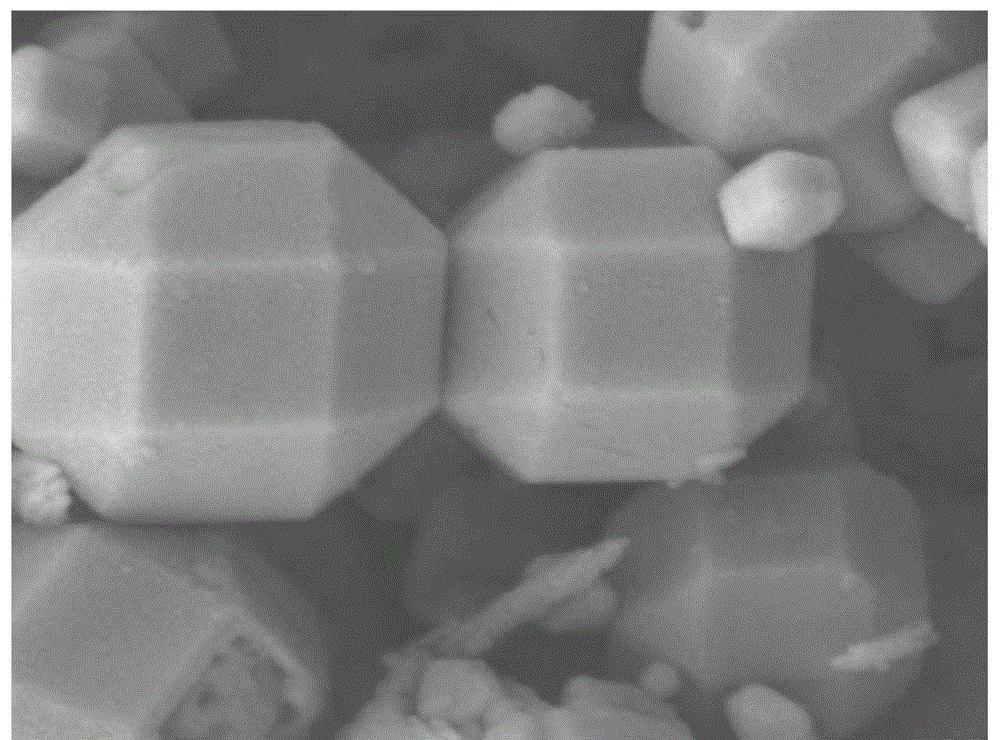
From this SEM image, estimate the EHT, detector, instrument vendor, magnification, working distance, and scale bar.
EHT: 5 kV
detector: LEI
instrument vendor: JEOL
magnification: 50 K X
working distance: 7.9 mm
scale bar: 100 nm